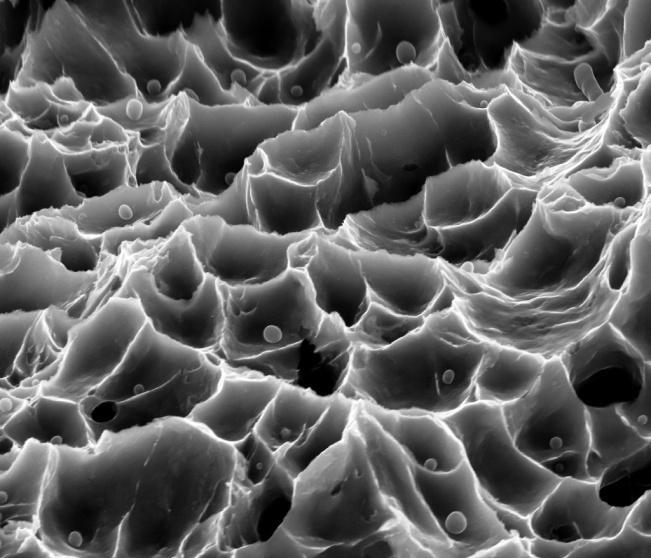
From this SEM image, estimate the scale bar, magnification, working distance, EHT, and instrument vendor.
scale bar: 10000 nm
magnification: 10.925 K X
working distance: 30.6 mm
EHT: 30 kV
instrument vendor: FEI